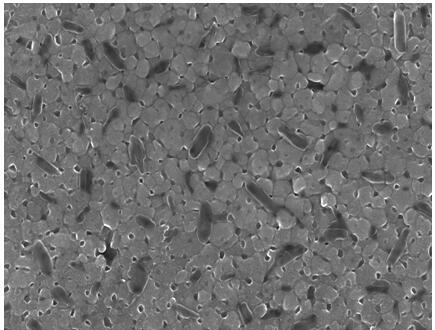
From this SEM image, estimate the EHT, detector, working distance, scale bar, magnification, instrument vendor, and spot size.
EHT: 15 kV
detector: TLD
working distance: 5.2 mm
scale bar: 1000 nm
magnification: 100 K X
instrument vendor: FEI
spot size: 3